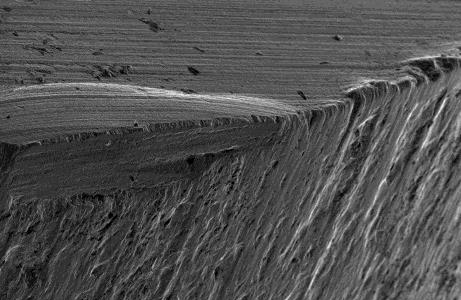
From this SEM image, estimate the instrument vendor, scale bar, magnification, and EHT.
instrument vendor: JEOL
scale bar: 100000 nm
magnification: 0.1 K X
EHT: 15 kV